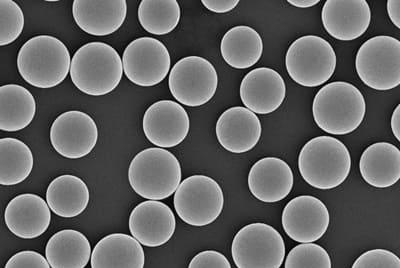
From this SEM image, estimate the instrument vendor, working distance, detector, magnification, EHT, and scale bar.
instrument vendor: Zeiss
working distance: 5.8 mm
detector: InLens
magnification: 0.3 K X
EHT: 5 kV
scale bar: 20000 nm